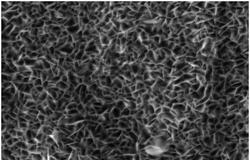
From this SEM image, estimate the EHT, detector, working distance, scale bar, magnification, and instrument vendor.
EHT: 2 kV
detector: SE2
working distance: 4.8 mm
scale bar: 200 nm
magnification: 30 K X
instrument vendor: Zeiss